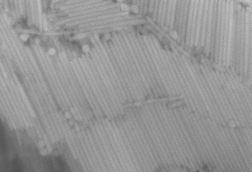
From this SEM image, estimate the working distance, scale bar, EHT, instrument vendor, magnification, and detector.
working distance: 13.1 mm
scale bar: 500 nm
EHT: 15 kV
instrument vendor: Hitachi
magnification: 100 K X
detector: SE(M)